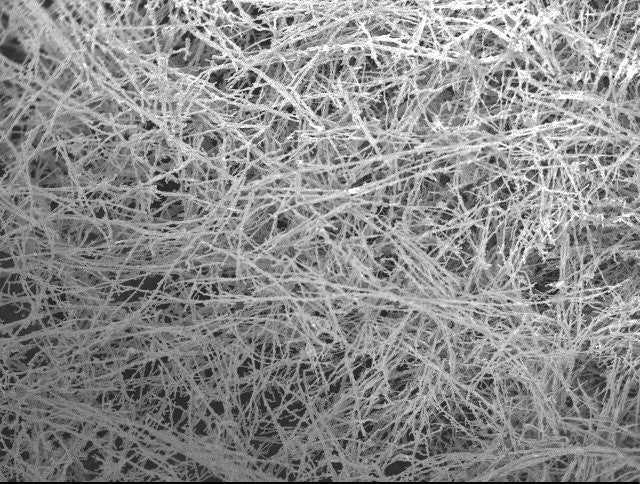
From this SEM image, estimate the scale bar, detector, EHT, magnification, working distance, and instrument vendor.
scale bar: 100000 nm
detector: SEI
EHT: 10 kV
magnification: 0.25 K X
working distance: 14.4 mm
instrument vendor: JEOL